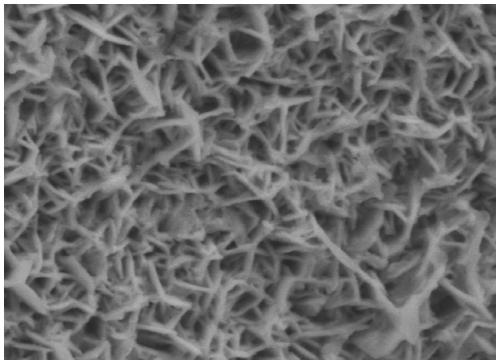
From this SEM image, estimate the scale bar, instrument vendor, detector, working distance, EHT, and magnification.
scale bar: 100 nm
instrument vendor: JEOL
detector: LED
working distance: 6.1 mm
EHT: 5 kV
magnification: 30 K X